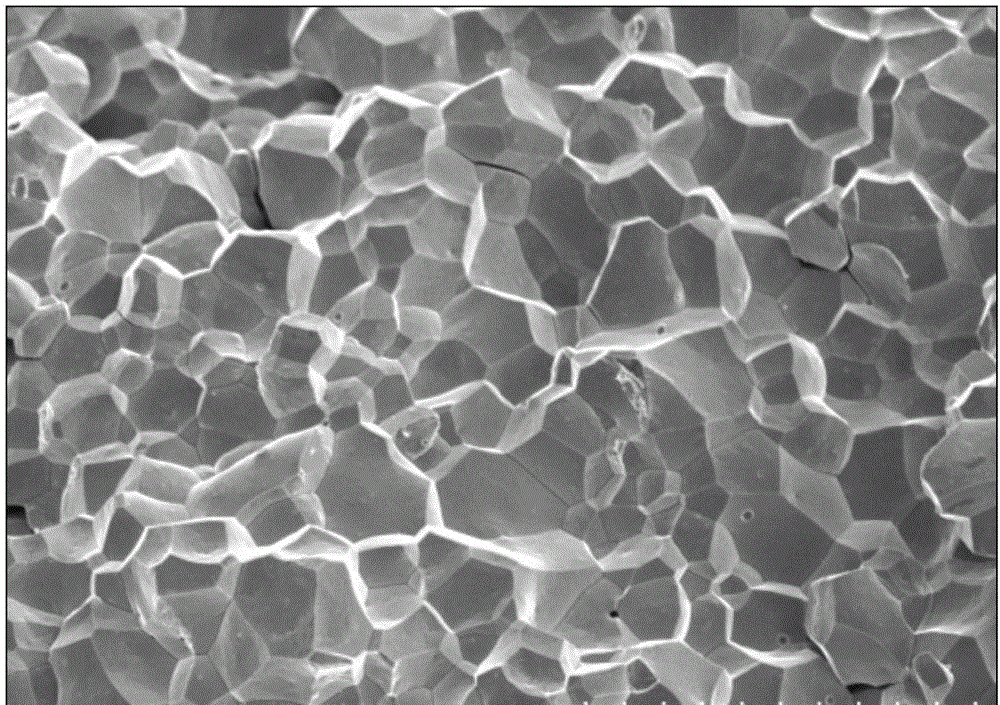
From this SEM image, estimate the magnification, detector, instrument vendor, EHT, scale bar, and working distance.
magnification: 0.5 K X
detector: SE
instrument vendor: Hitachi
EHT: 20 kV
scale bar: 100000 nm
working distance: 10.1 mm